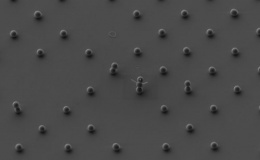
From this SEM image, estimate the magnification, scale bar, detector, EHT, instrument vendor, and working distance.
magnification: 3 K X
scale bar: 2000 nm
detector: SE2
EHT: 3 kV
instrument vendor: Zeiss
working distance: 4.4 mm